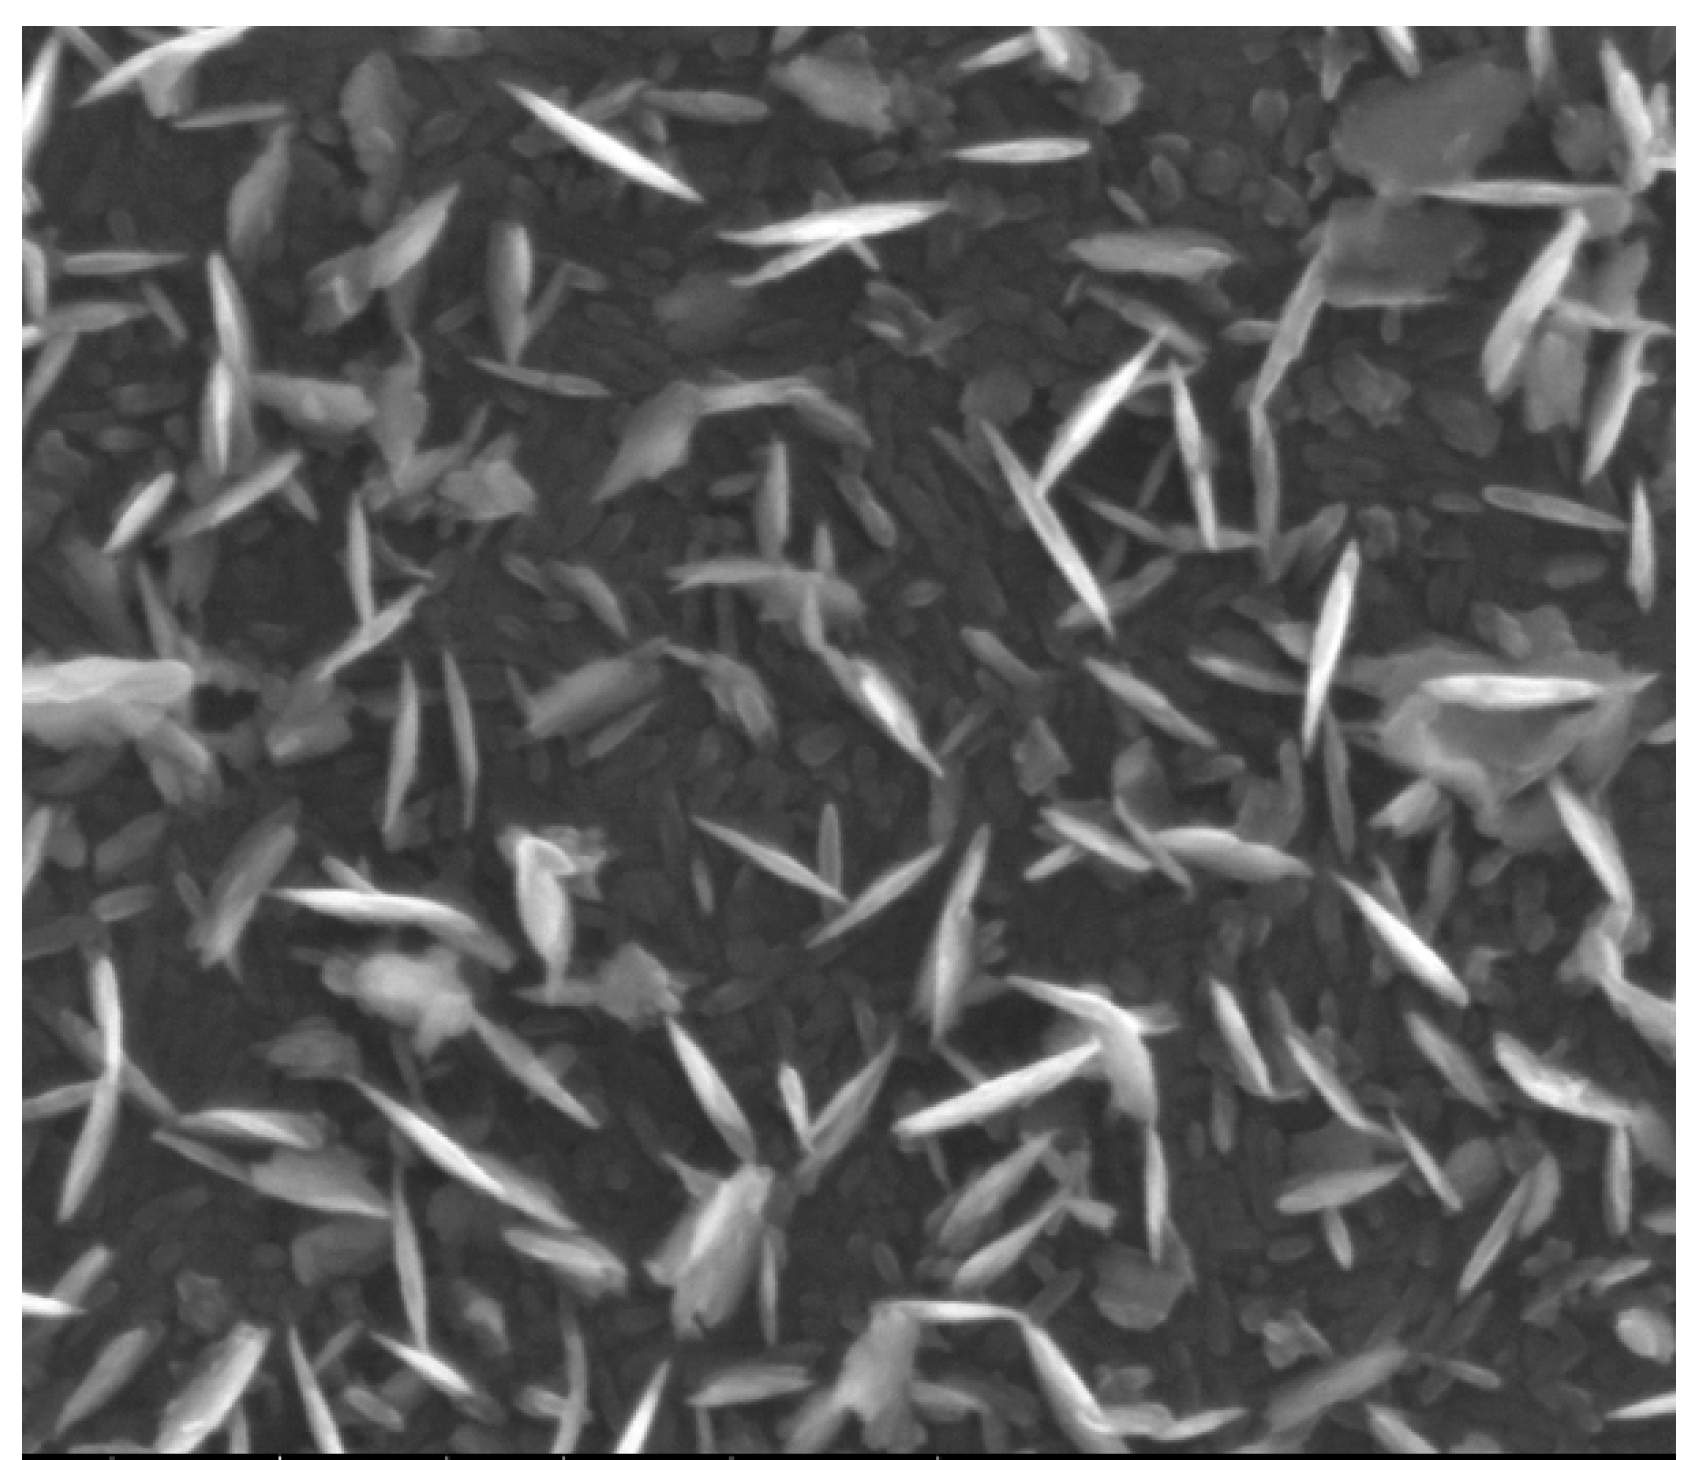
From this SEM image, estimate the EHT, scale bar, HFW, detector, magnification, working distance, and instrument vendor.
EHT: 10 kV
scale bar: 1000 nm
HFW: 2.84 µm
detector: Helix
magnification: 100 K X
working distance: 5.4 mm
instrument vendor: FEI